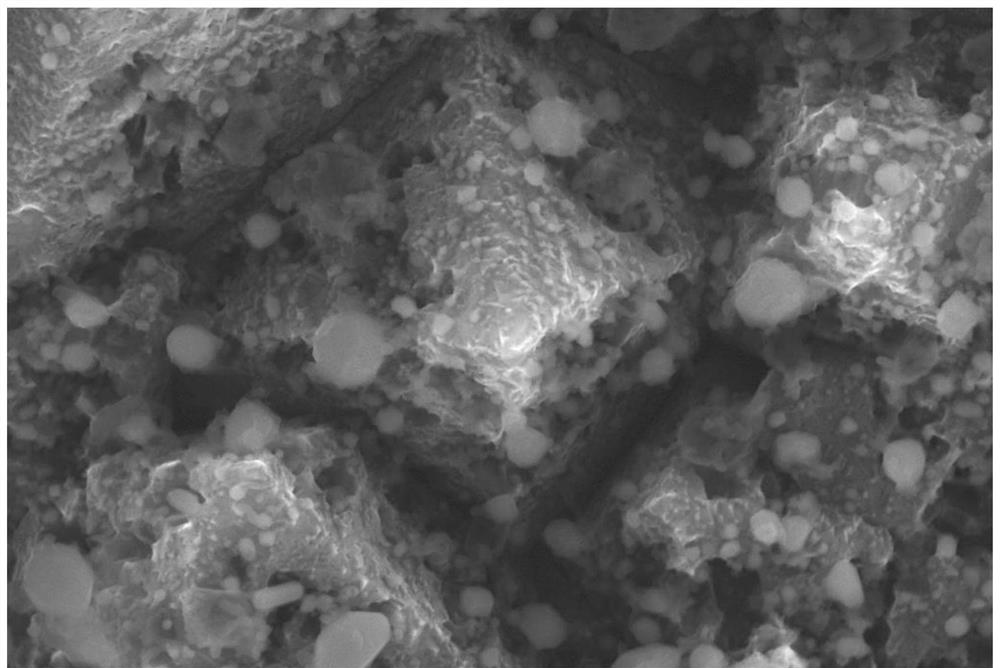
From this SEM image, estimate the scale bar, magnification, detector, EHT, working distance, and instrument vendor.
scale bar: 200 nm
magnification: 30 K X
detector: InLens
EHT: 10 kV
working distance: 2 mm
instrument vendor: Zeiss